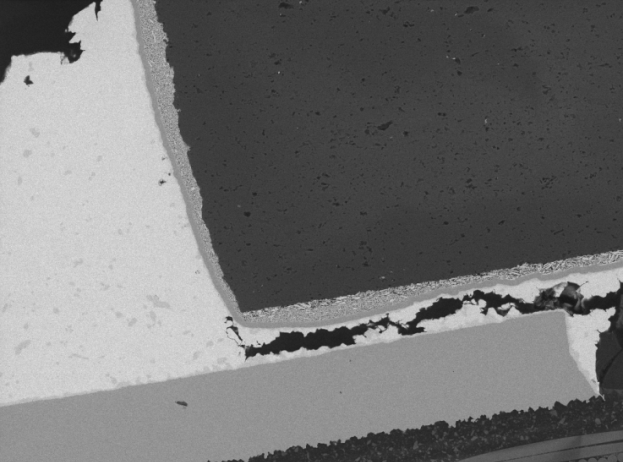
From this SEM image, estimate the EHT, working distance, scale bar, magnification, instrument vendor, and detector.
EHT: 20 kV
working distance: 15.11 mm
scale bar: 100000 nm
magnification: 0.505 K X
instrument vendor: Tescan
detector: BSE